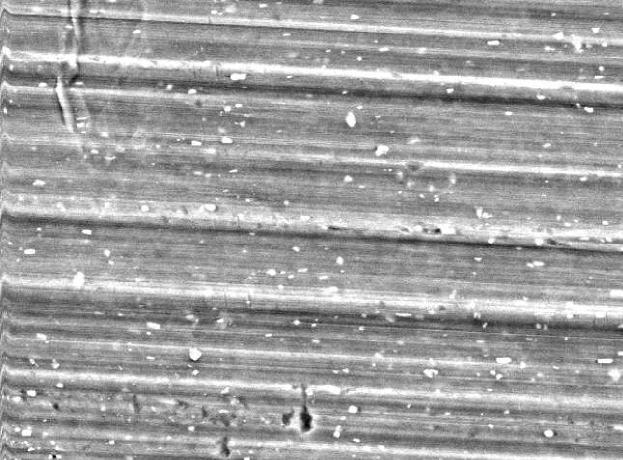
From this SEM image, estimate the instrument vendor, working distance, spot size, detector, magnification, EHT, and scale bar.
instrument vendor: FEI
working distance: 9.3 mm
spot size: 6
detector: SE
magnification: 0.786 K X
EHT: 20 kV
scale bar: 20000 nm